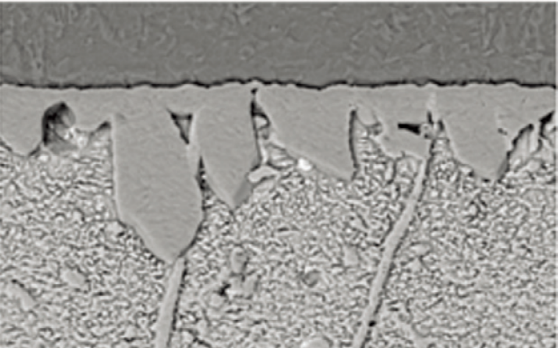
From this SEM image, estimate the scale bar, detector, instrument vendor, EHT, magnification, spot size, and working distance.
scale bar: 10000 nm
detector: BSE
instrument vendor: FEI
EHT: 15 kV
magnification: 2 K X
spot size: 3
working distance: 10.7 mm